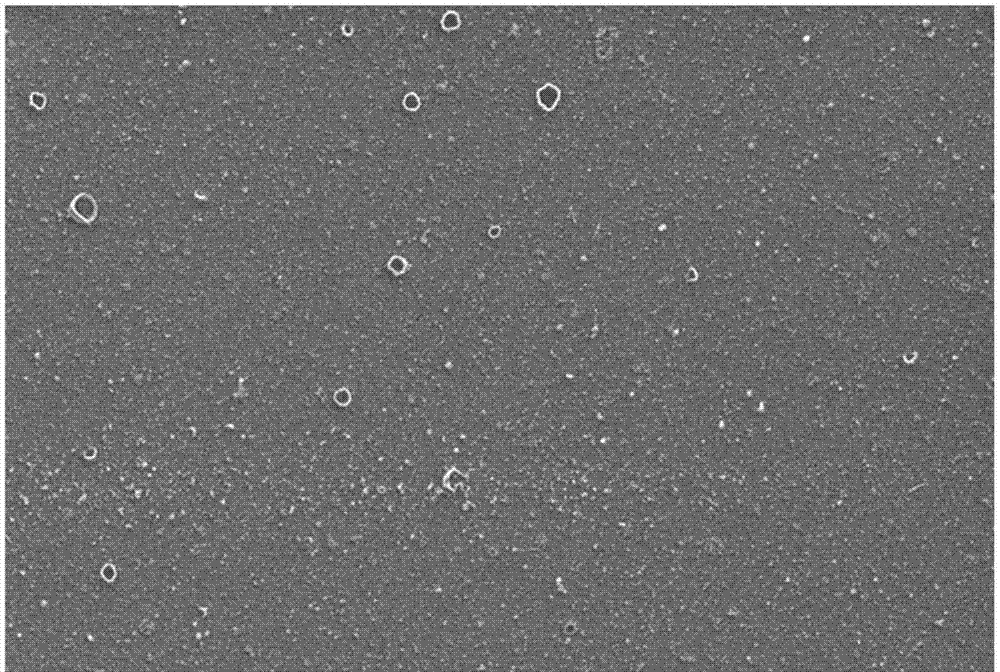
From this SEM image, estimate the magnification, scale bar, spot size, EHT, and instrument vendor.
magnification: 5 K X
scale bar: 5000 nm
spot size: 3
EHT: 10 kV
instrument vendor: FEI